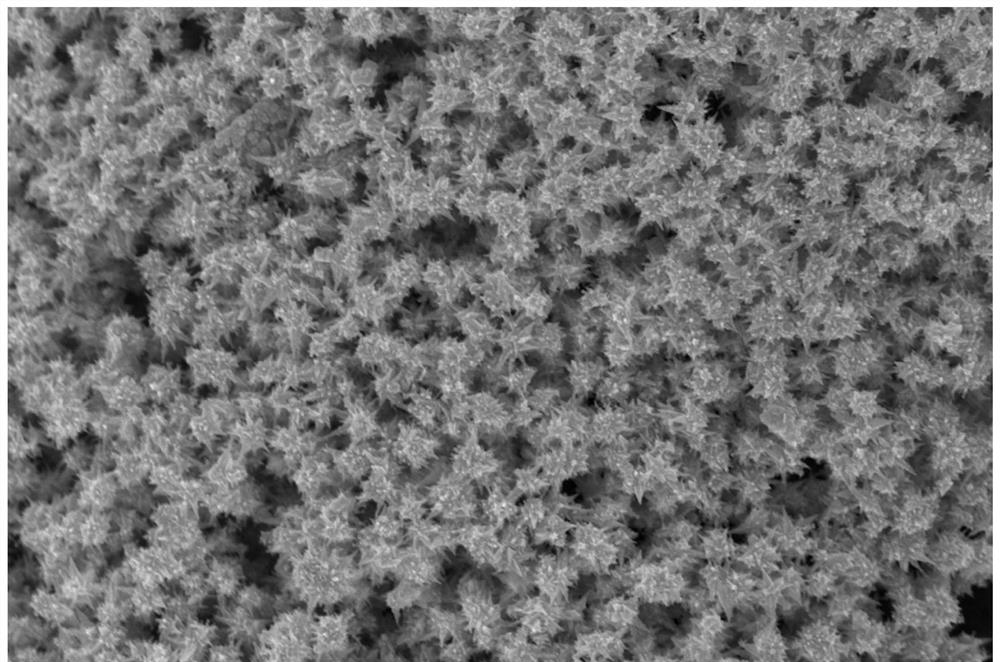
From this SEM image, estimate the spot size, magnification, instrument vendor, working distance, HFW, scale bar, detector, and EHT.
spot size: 3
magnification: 30 K X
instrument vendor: FEI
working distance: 4.8 mm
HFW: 6.91 µm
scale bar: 1000 nm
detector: TLD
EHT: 15 kV